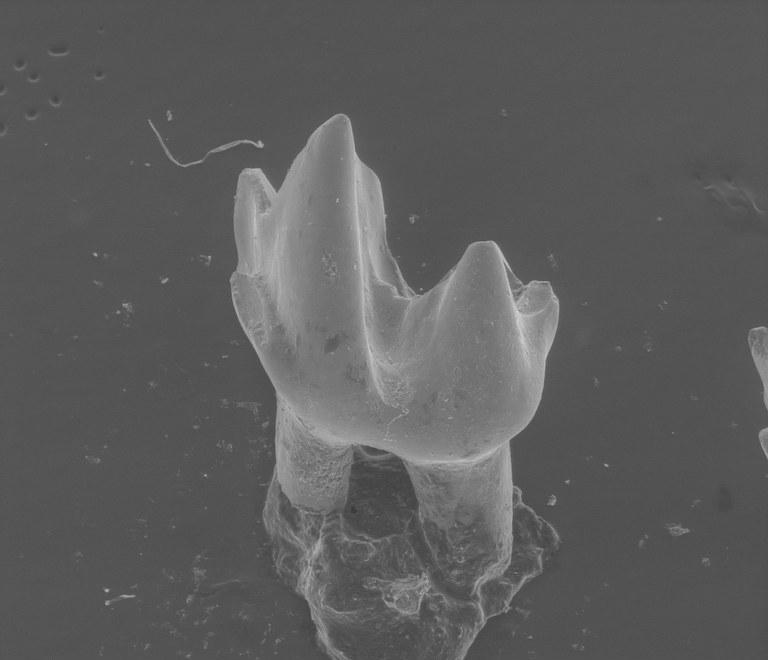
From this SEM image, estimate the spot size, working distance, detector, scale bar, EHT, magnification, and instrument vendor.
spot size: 4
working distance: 33.2 mm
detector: LFD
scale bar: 1000000 nm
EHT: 20 kV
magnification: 0.09 K X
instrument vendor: FEI